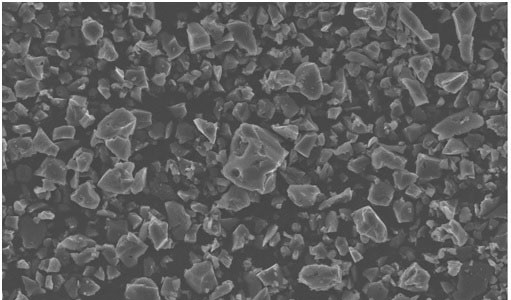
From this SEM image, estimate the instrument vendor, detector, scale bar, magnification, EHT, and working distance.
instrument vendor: Zeiss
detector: InLens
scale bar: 10000 nm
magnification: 1 K X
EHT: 10 kV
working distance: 3.7 mm